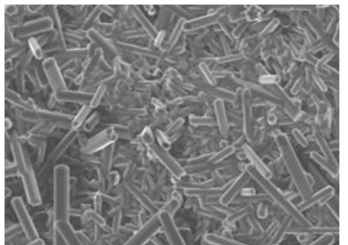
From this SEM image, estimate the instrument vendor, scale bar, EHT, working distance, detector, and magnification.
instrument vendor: JEOL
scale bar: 10000 nm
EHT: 15 kV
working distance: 17 mm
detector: SEI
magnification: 1 K X